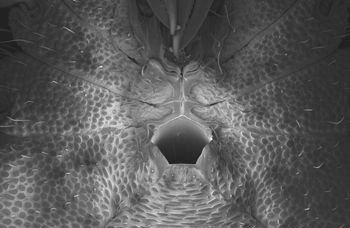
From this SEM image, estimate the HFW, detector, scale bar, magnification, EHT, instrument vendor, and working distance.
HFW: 946.2 µm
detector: InLens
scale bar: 100000 nm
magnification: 0.32 K X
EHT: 5 kV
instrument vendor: Zeiss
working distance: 12.2 mm